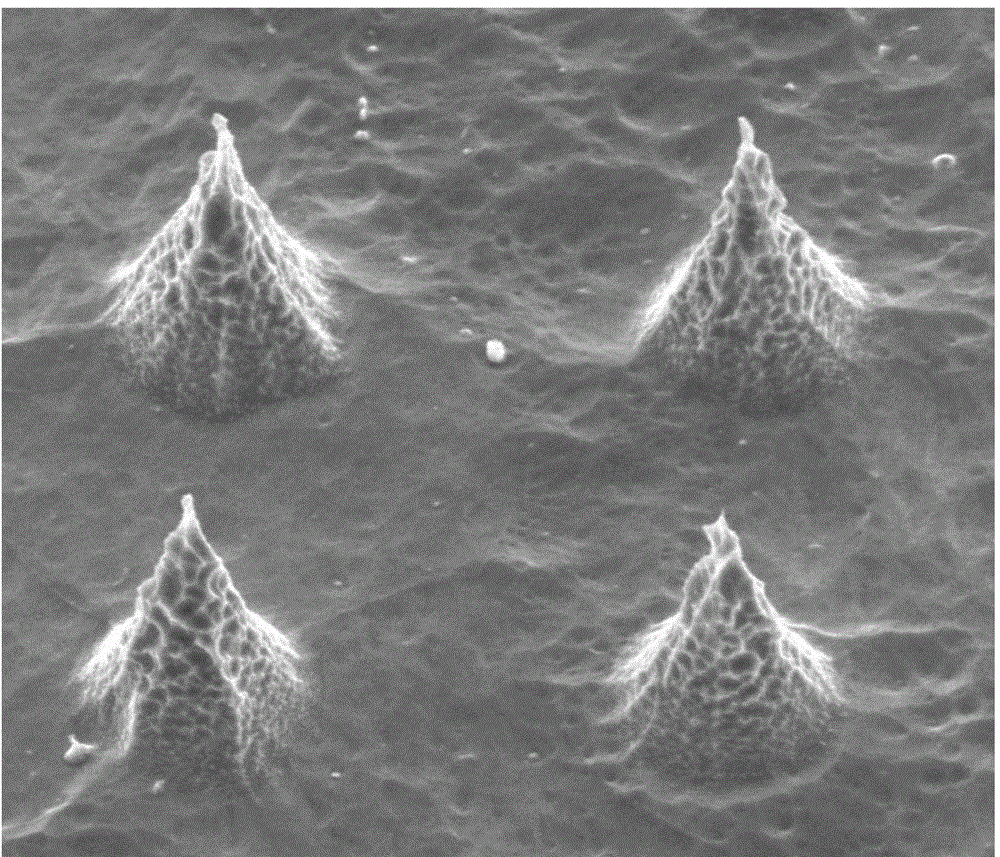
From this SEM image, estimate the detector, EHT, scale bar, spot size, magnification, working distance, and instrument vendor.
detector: ETD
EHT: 10 kV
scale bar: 5000 nm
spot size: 2.5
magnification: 16 K X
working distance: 5.6 mm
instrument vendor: FEI